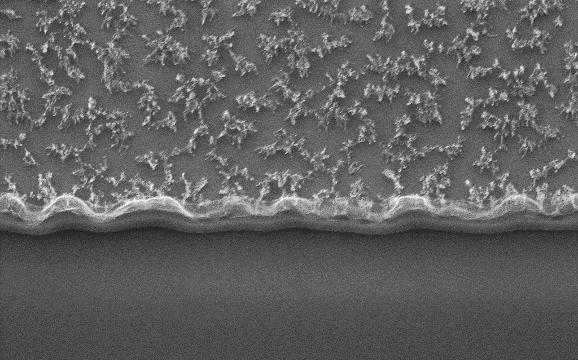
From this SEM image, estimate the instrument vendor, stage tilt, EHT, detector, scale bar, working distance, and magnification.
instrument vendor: Zeiss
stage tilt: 20°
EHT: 15 kV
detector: SE2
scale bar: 1000 nm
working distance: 16.6 mm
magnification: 20 K X